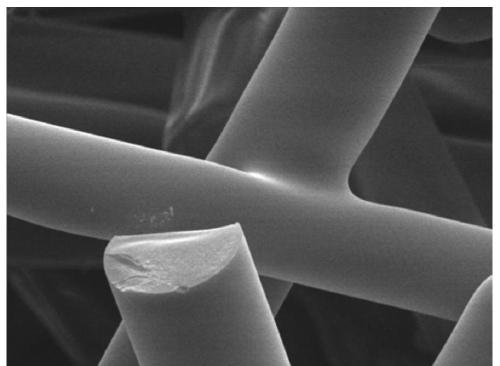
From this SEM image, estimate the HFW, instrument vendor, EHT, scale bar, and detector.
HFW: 48 µm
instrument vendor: JEOL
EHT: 15 kV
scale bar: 10000 nm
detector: SEI